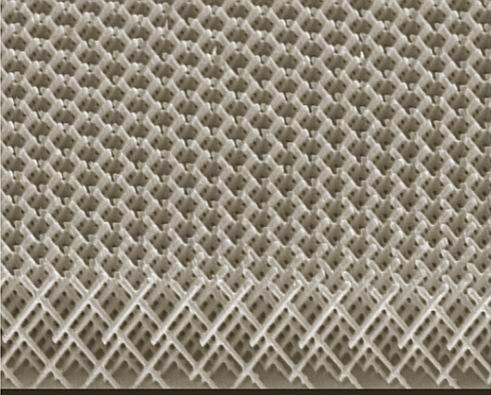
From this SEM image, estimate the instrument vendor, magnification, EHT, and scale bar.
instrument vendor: JEOL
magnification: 2.2 K X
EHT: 10 kV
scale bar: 10000 nm